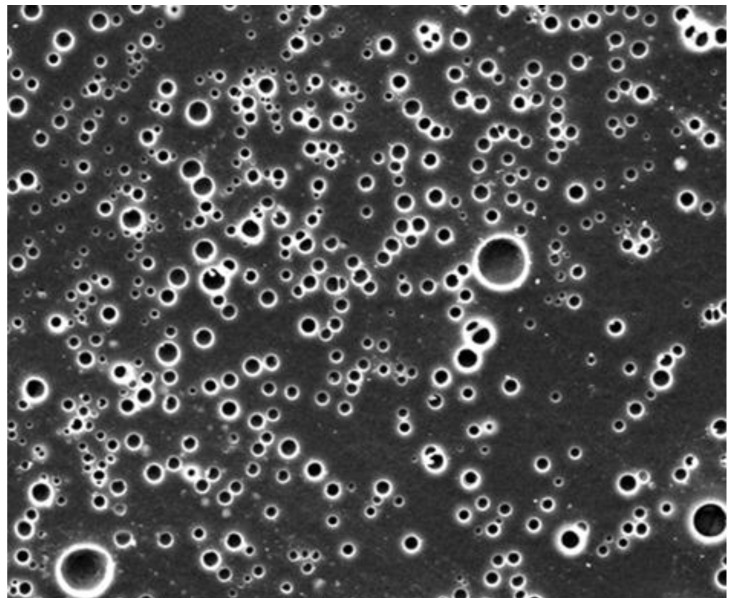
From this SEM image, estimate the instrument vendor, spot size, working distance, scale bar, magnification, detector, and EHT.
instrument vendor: FEI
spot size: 7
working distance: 25.1 mm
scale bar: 100000 nm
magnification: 1 K X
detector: ETD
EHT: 15 kV